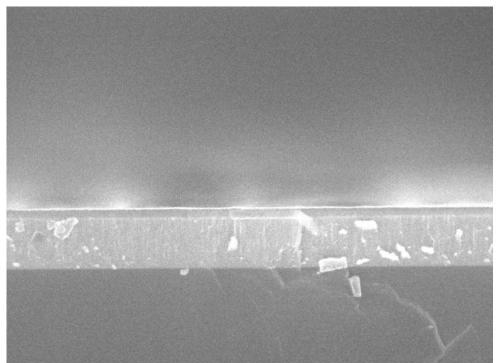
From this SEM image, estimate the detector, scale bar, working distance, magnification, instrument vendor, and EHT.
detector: SEI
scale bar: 100 nm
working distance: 7.3 mm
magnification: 30 K X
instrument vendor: JEOL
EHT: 15 kV